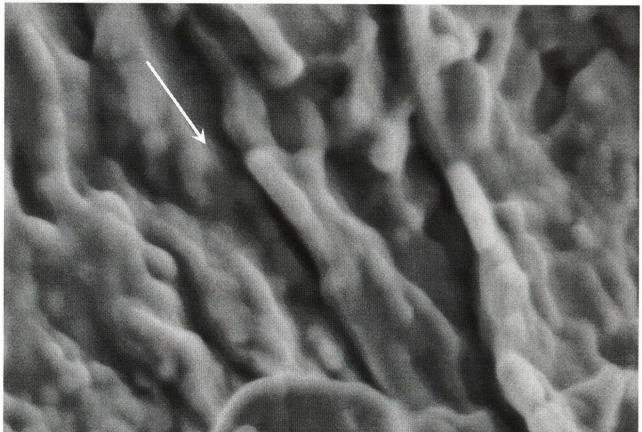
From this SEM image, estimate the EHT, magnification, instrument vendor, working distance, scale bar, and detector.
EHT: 1.3 kV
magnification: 509.76 K X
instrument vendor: Zeiss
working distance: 4.4 mm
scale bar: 100 nm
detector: InLens